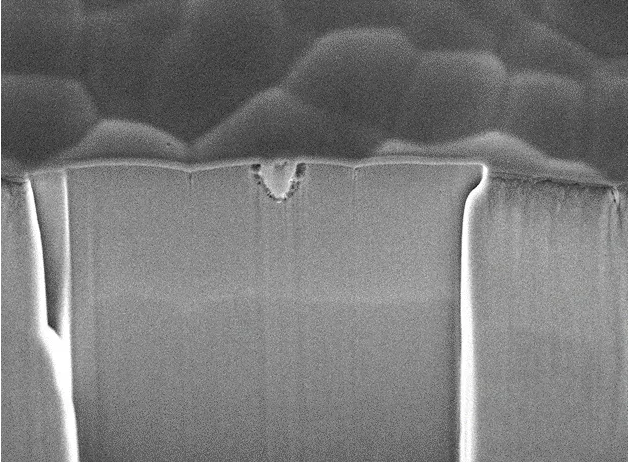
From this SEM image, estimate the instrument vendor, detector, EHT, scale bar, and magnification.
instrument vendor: JEOL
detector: SEI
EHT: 10 kV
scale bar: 1000 nm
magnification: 7 K X